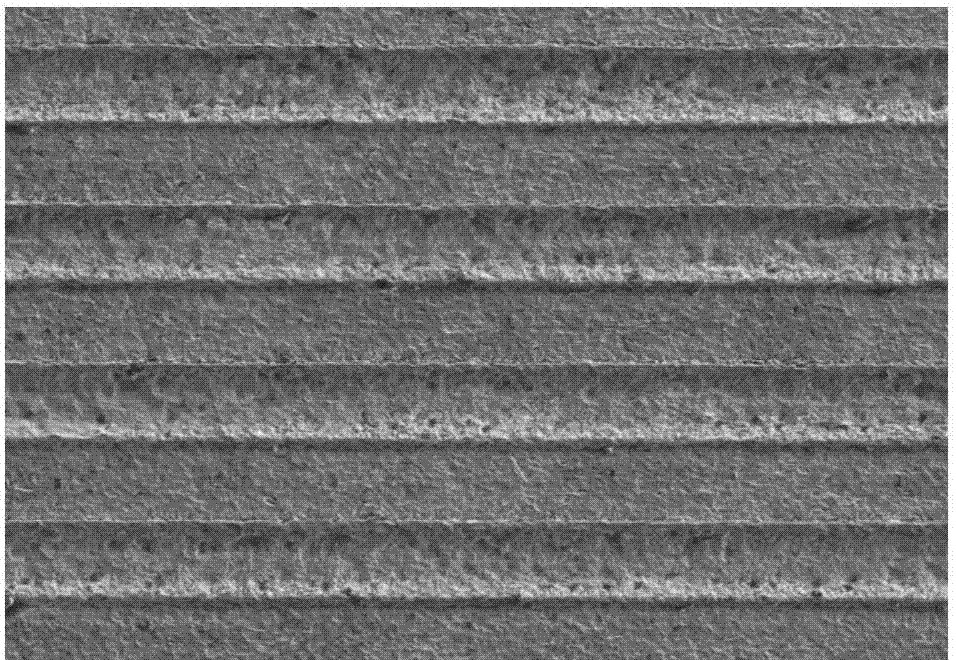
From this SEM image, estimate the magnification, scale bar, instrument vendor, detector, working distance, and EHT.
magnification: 0.1 K X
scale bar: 500000 nm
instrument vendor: Hitachi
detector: LM(UL)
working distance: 7.9 mm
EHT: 10 kV